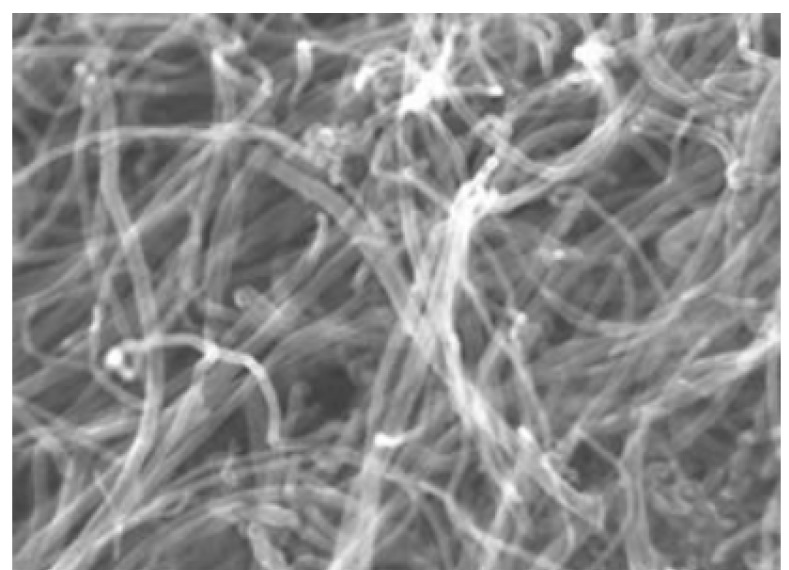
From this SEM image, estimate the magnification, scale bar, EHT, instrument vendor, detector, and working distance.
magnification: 100 K X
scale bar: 500 nm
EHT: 15 kV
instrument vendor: Hitachi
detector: SE(M)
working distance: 8.8 mm